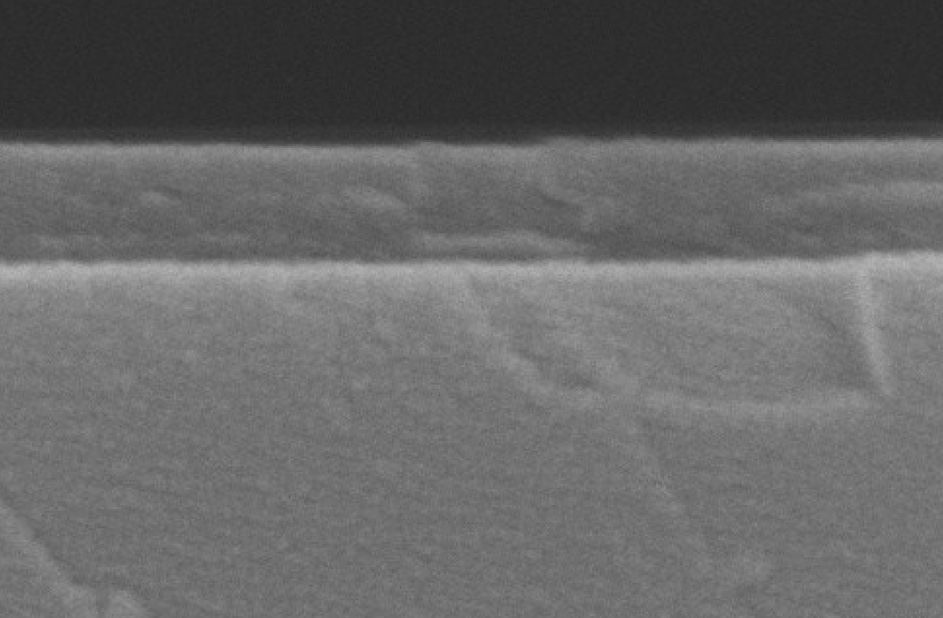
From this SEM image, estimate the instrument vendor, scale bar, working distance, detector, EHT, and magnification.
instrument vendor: JEOL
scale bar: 100 nm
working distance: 7.4 mm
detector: SEI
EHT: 10 kV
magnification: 200 K X